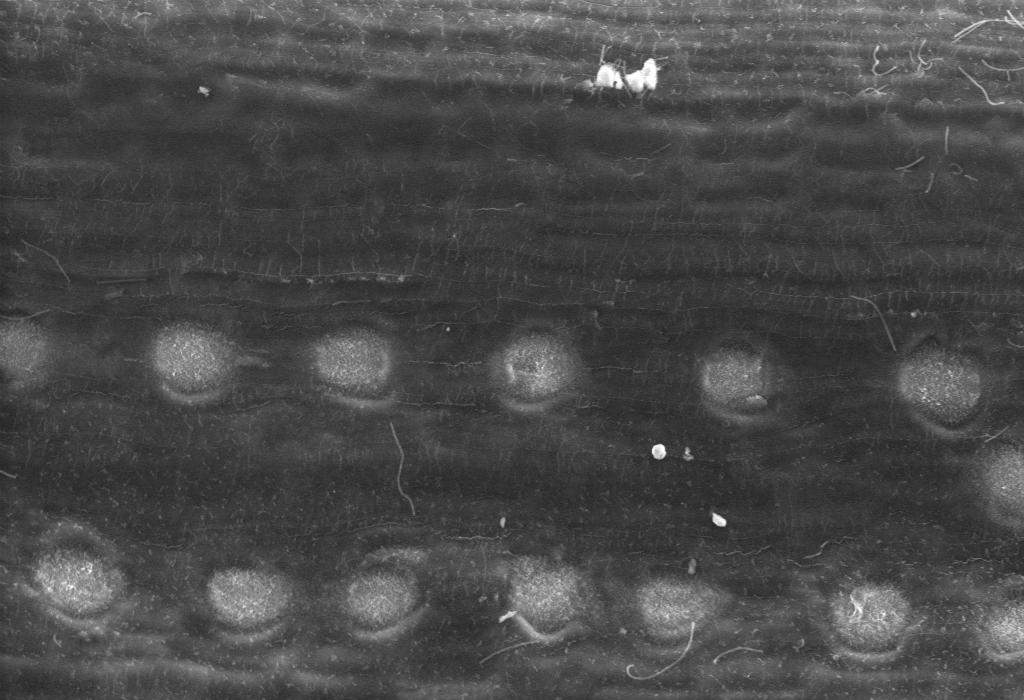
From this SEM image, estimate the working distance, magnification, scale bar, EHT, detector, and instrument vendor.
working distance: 10 mm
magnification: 0.707 K X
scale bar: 100000 nm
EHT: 20 kV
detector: VPSE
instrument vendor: Zeiss